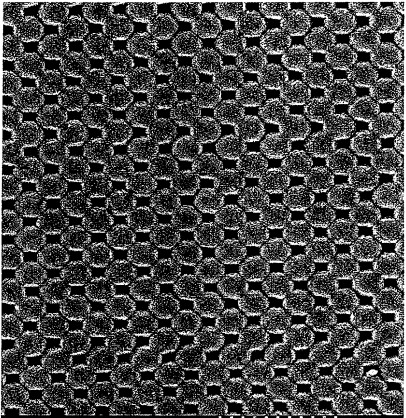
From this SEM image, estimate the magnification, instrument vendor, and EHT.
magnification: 30 K X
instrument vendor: Hitachi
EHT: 5 kV